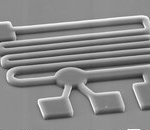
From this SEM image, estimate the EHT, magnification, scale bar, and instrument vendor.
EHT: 20 kV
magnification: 0.65 K X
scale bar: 10000 nm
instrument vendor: JEOL